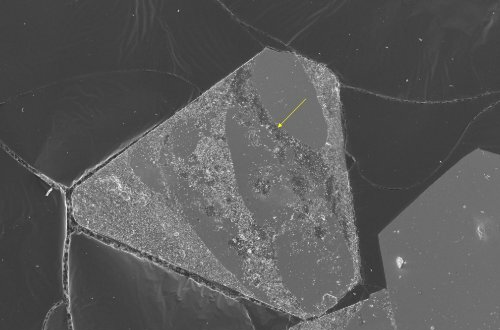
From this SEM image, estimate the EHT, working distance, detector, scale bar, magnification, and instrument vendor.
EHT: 3 kV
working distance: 4 mm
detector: InLens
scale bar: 10000 nm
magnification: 1.14 K X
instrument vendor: Zeiss